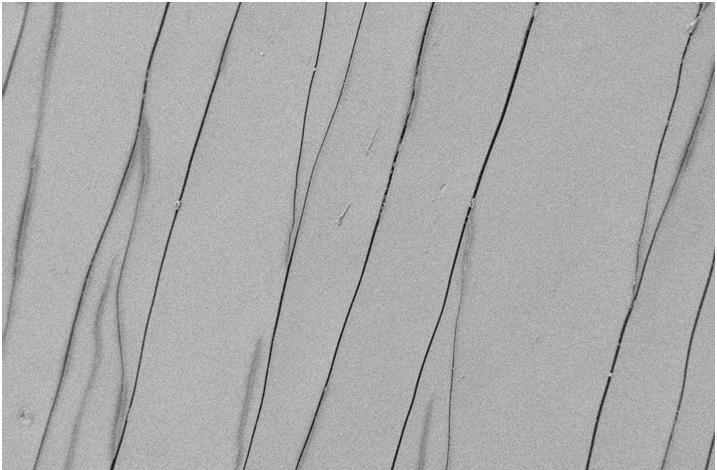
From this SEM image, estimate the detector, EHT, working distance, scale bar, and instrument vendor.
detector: SE2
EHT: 5 kV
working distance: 7.3 mm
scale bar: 10000 nm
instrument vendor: Zeiss